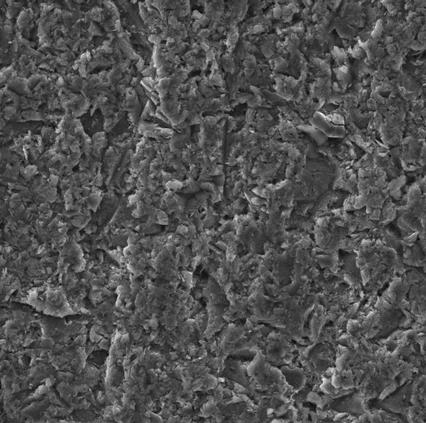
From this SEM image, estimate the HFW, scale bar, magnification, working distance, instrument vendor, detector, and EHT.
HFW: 173 µm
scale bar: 50000 nm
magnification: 1.6 K X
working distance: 14.93 mm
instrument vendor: Tescan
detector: SE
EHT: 20 kV